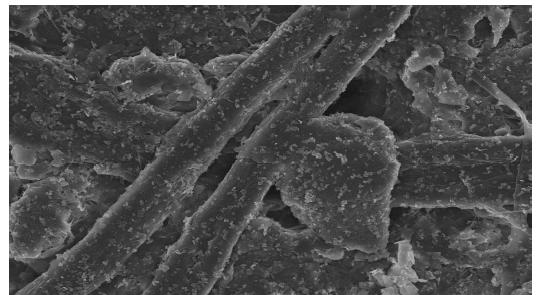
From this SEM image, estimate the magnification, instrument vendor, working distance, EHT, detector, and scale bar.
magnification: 1 K X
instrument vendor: Hitachi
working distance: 12 mm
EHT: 15 kV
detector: SE(U)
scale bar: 50000 nm